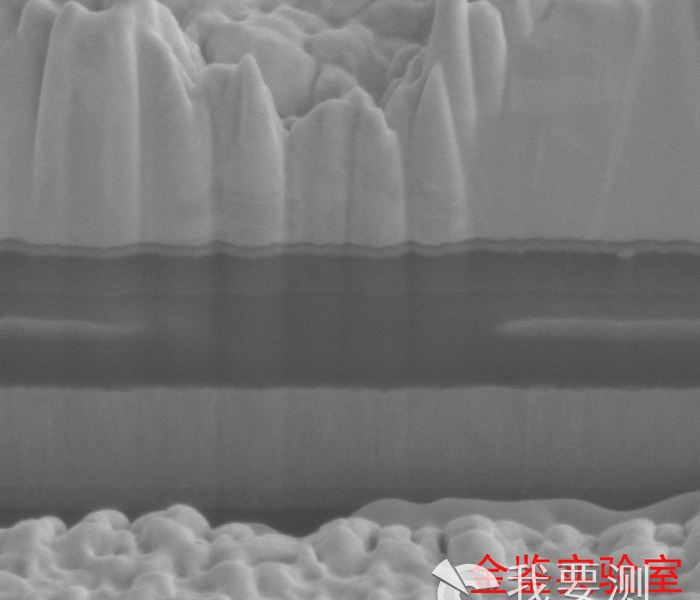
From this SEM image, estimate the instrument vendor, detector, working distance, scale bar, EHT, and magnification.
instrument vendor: FEI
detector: ETD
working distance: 4.9 mm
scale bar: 1000 nm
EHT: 5 kV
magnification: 60 K X